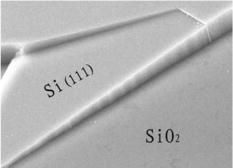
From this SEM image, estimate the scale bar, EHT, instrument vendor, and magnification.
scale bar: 10000 nm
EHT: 25 kV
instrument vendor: other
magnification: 2.28 K X